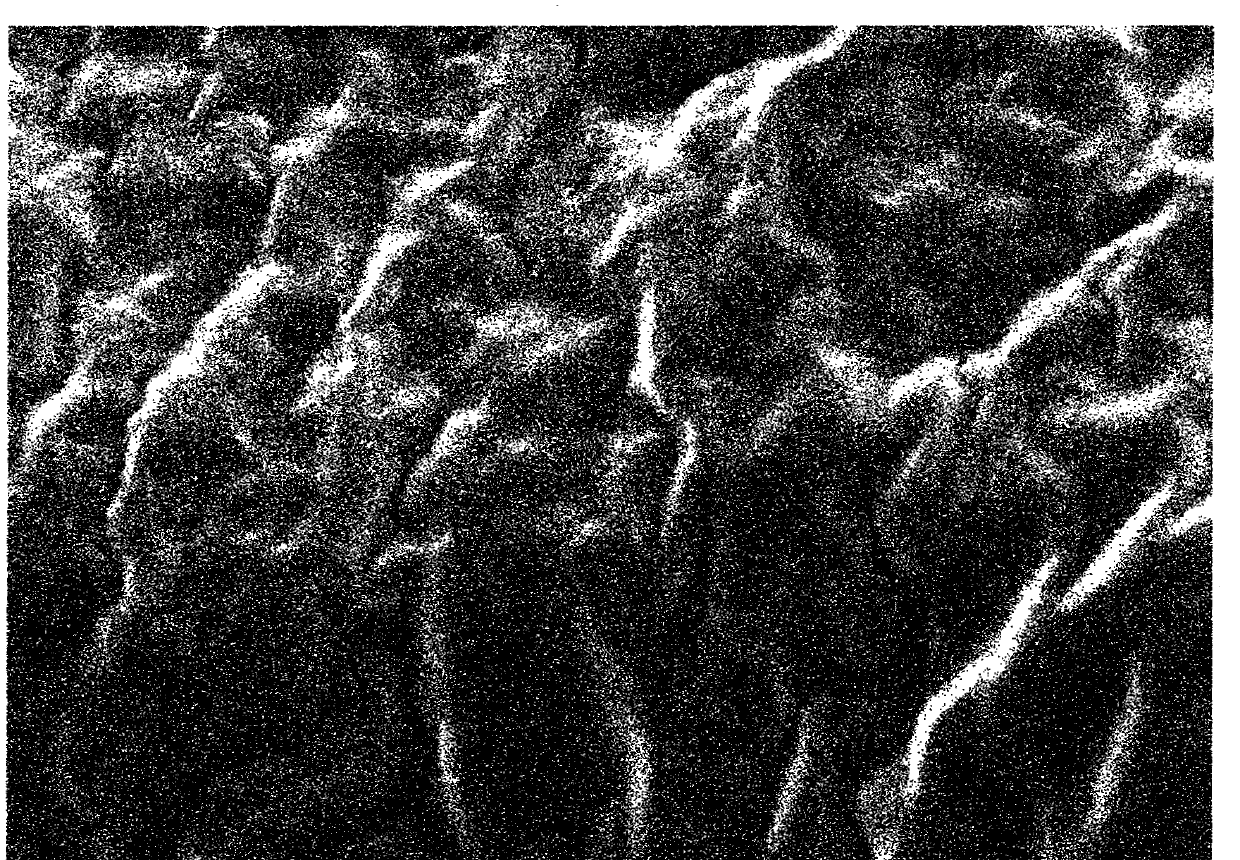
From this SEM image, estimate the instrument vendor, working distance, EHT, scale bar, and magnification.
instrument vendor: Hitachi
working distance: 10.2 mm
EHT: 10 kV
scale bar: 2000 nm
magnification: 5 K X